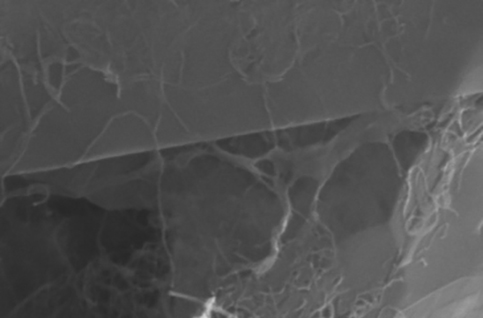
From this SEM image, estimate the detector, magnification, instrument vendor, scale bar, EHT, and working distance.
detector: SE2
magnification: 500 K X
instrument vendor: Zeiss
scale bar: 100 nm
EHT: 20 kV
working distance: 9.9 mm